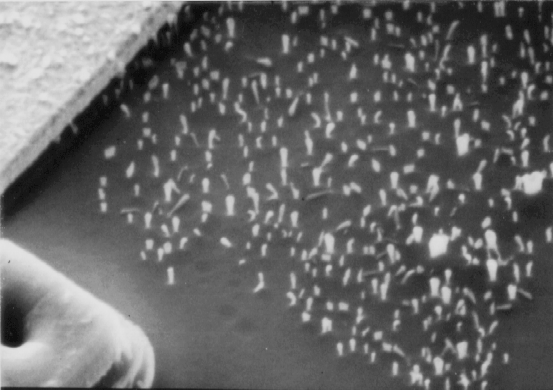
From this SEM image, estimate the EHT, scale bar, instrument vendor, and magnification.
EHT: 10 kV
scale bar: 1000 nm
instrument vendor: other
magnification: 10 K X